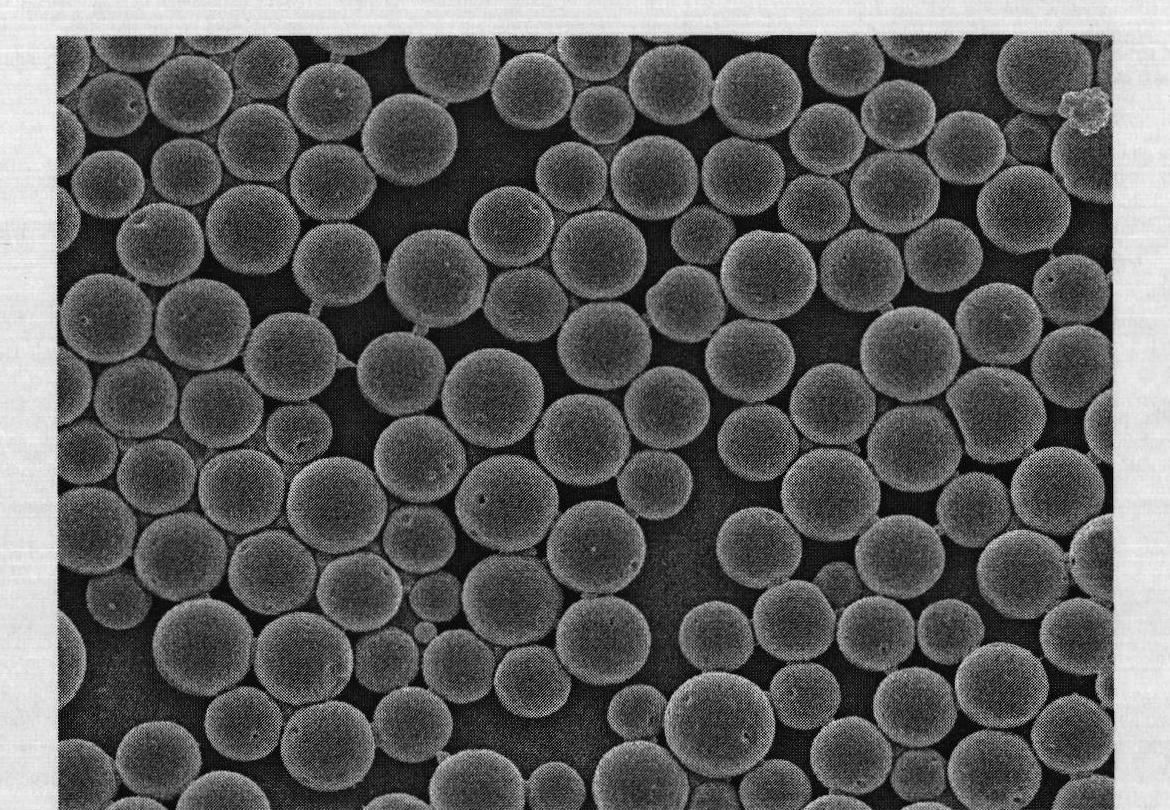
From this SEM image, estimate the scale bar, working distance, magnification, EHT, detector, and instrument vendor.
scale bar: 1000 nm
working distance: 6.7 mm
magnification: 5 K X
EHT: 5 kV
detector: SEI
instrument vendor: JEOL